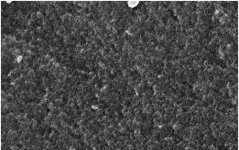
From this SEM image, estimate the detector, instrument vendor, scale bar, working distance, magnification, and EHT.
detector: InLens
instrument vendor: Zeiss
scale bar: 100 nm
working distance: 2.2 mm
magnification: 250 K X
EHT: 5 kV